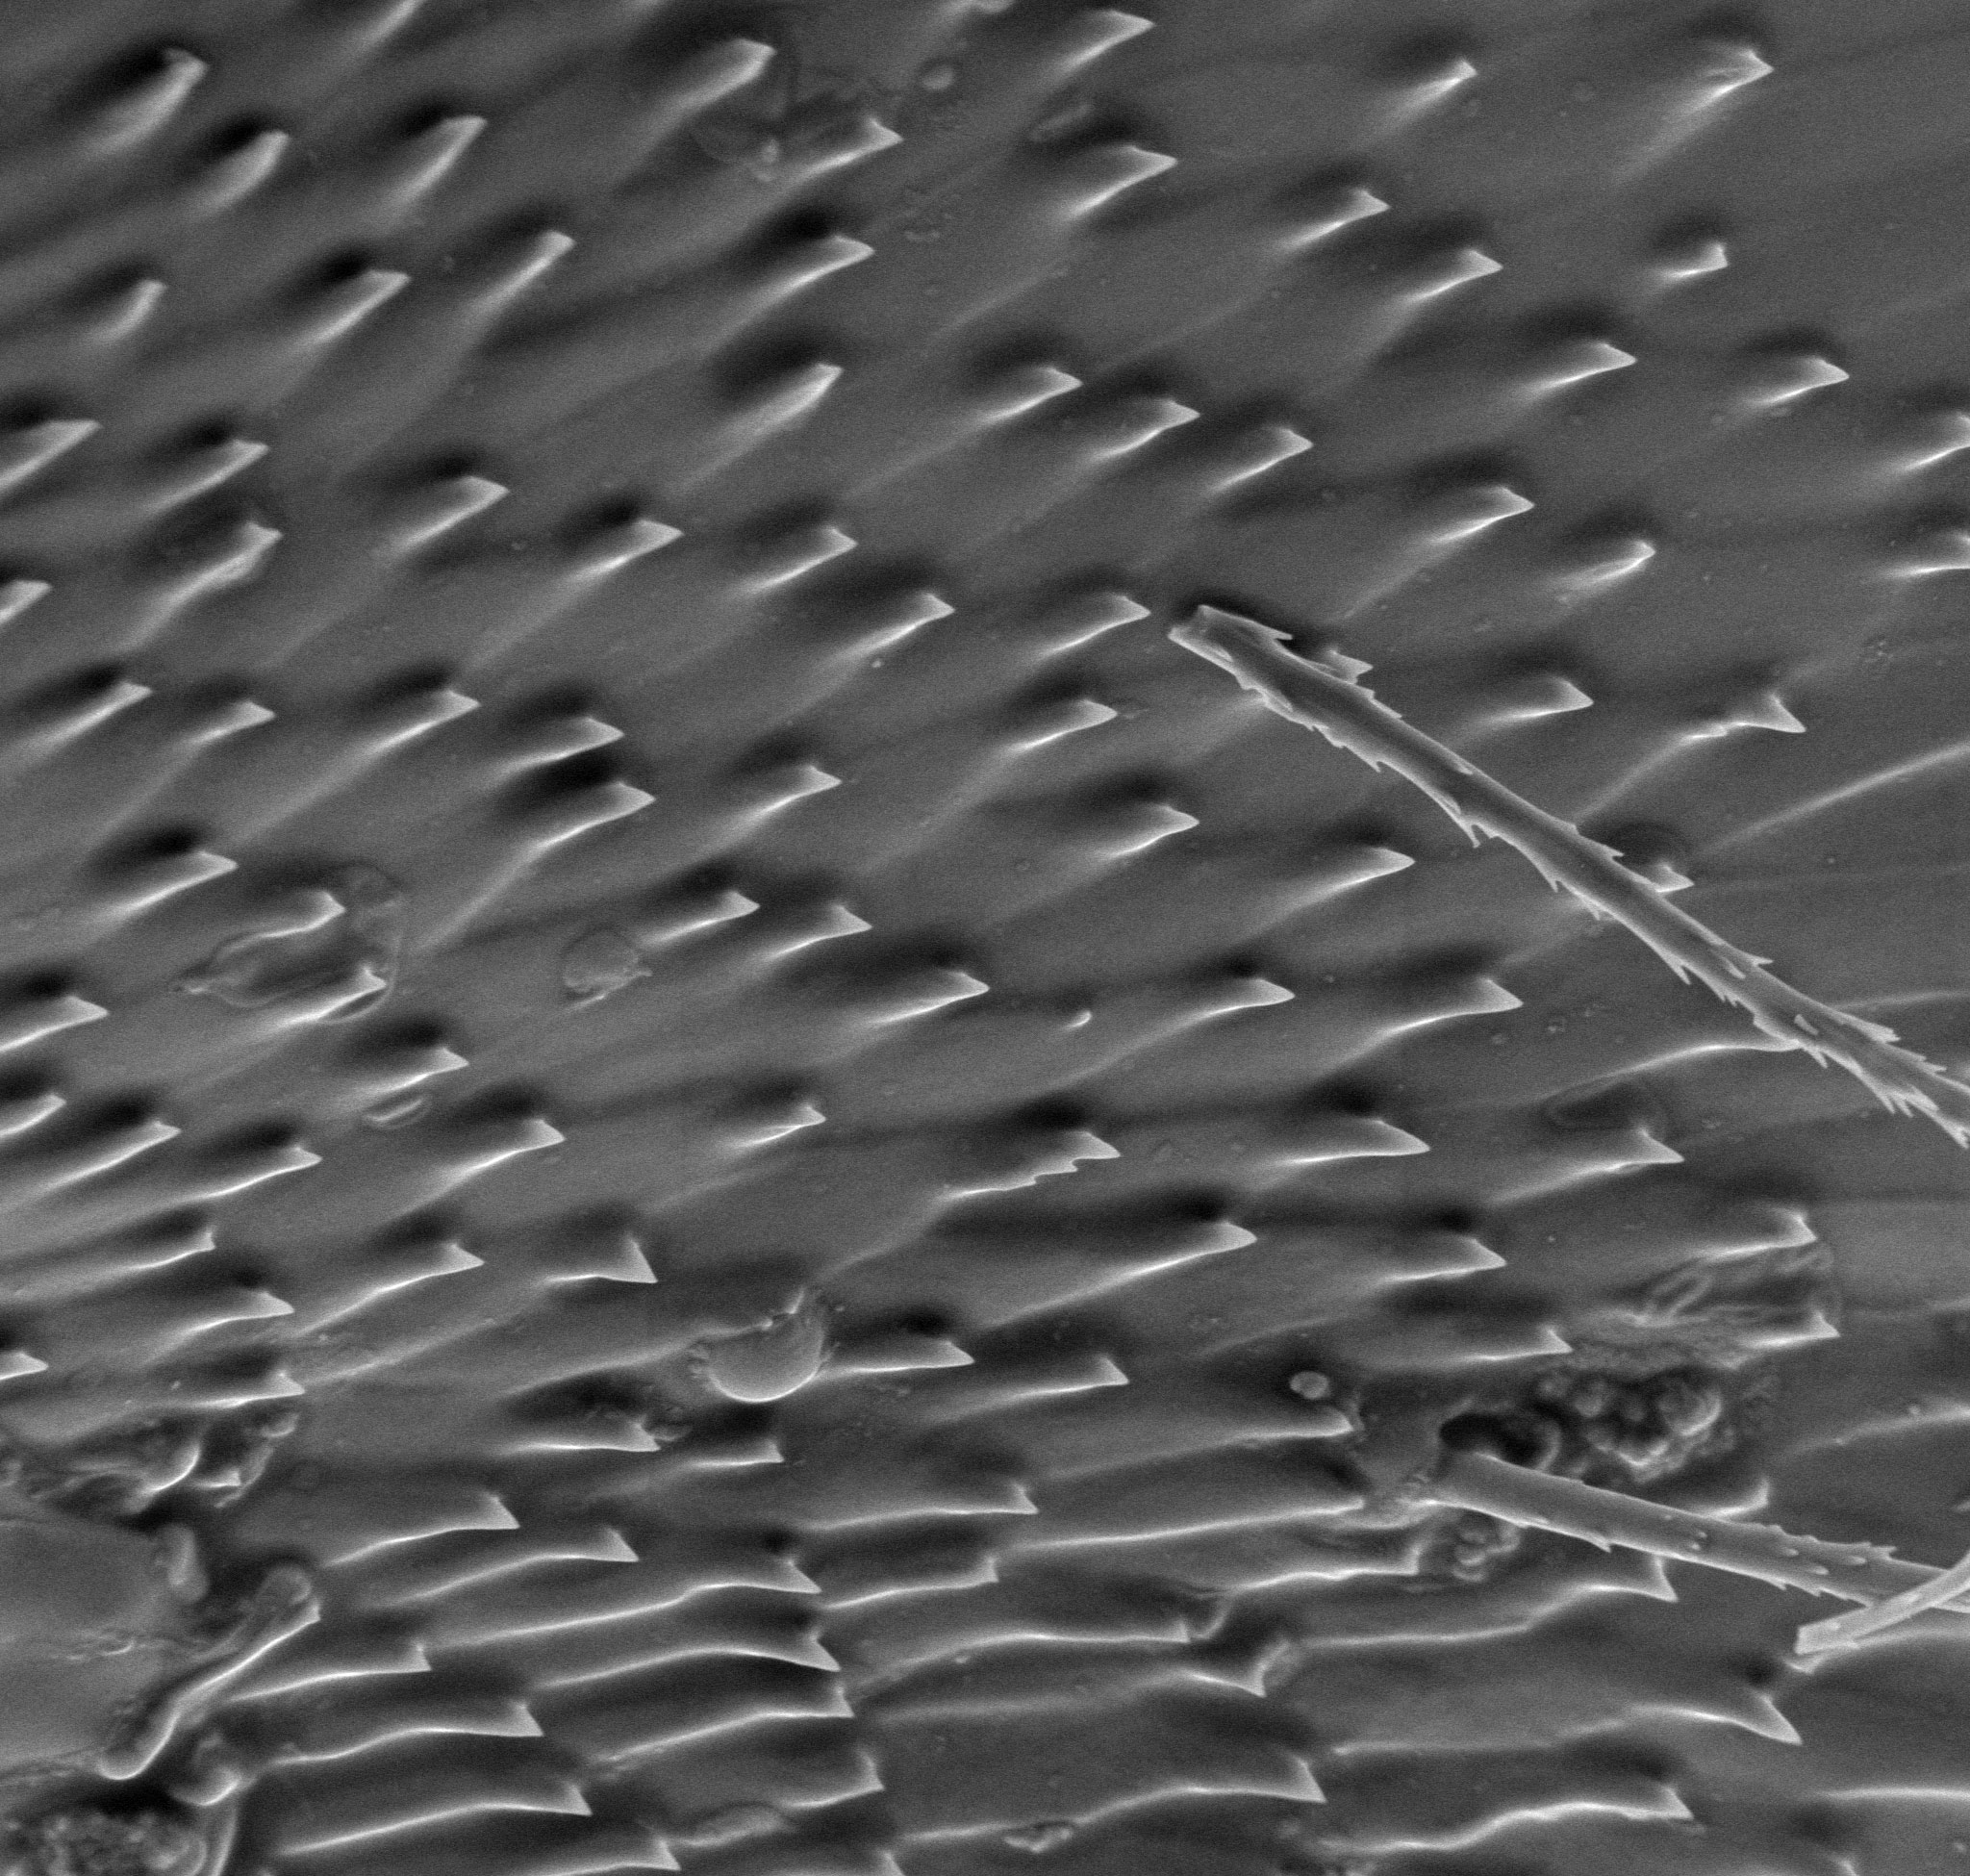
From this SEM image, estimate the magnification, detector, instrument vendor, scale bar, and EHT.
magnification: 1.87 K X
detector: SE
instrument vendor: other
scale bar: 20000 nm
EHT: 10 kV